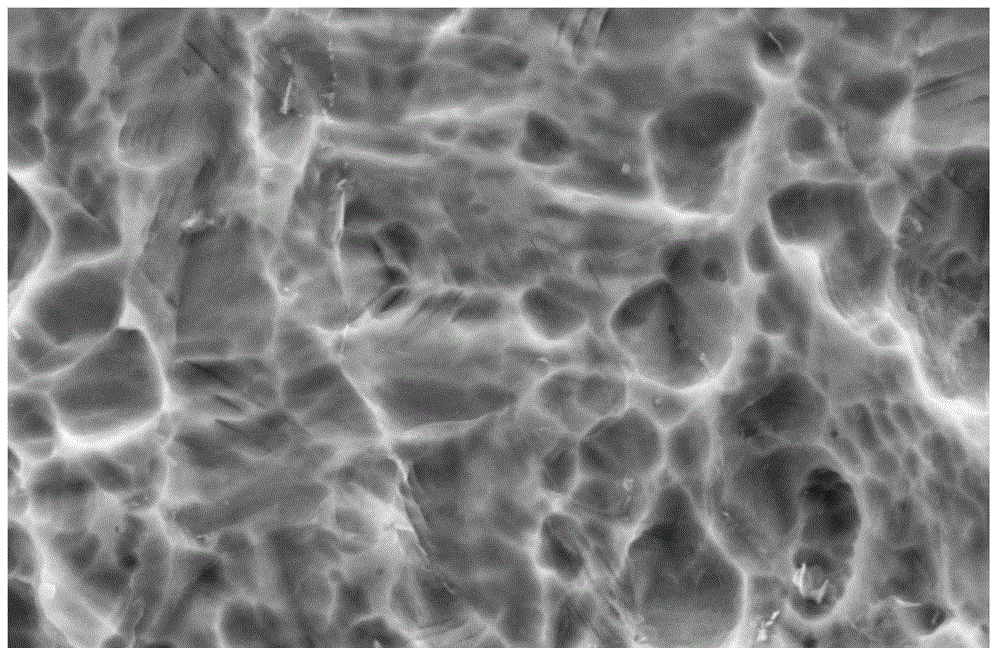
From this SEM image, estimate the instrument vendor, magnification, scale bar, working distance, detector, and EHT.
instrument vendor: Zeiss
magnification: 10 K X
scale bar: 2000 nm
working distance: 10.1 mm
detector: SE2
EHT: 20 kV